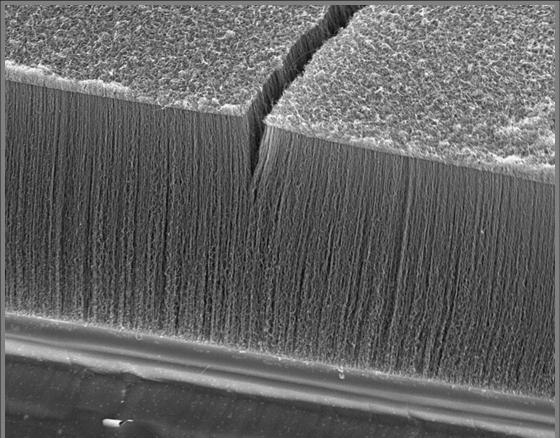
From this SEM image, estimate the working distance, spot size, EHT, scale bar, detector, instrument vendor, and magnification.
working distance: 6.6 mm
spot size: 4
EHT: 10 kV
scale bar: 10000 nm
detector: TLD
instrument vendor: FEI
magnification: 10 K X